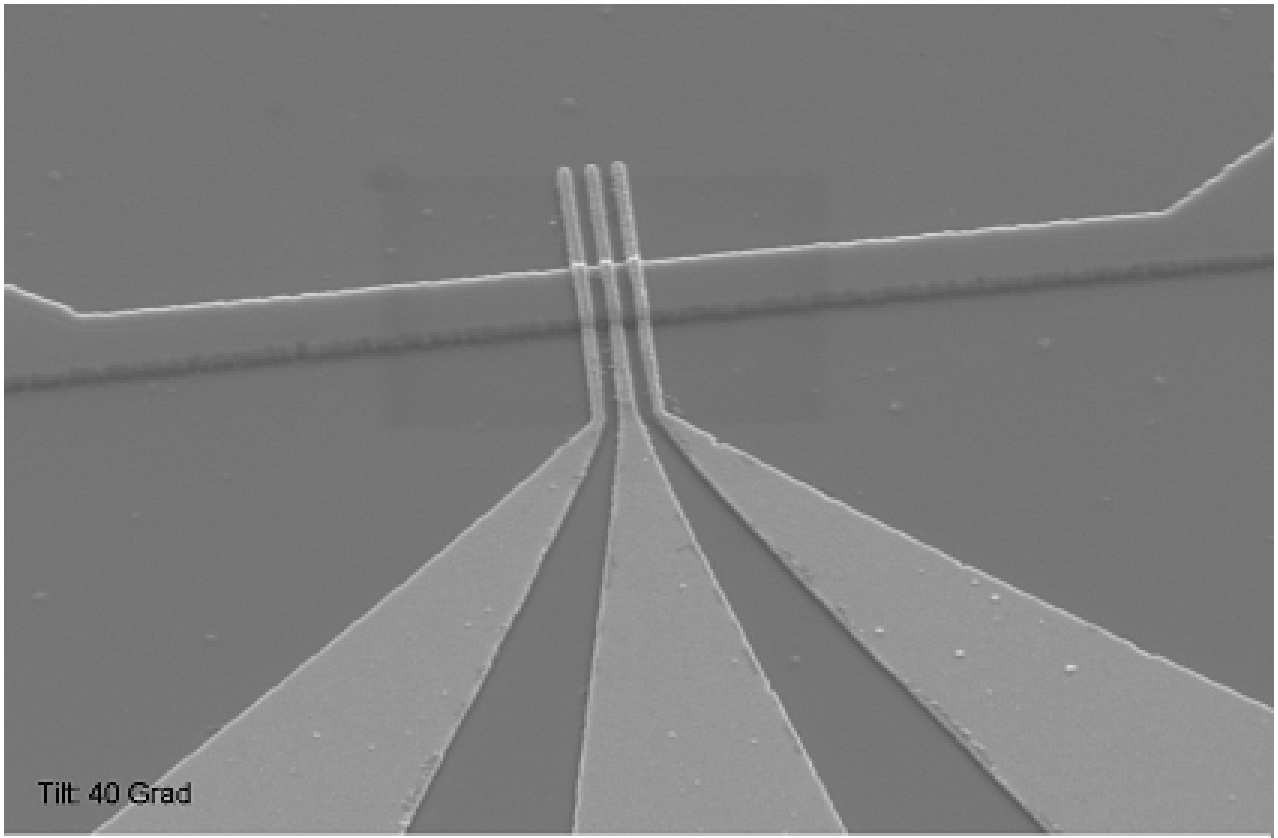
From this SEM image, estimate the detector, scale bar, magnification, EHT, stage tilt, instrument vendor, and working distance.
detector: SE2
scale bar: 1000 nm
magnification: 23.08 K X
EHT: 5 kV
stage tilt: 40°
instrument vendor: Zeiss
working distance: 13 mm